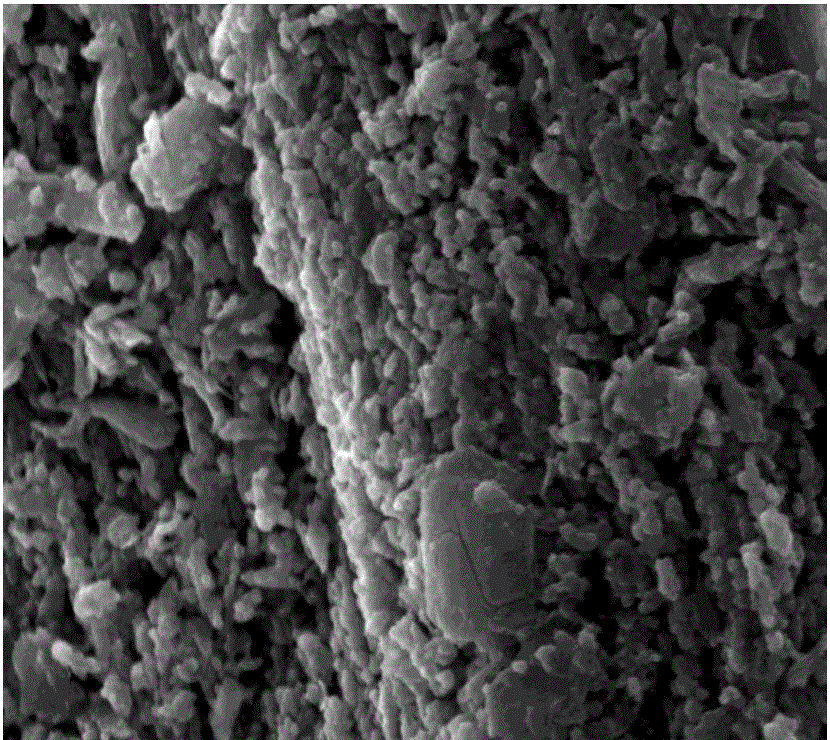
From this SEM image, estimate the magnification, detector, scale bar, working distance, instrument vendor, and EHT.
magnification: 20 K X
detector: SE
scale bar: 5000 nm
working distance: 10.5 mm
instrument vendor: FEI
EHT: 20 kV